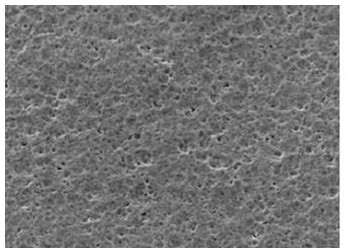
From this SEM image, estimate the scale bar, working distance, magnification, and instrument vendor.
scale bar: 50000 nm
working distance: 18.2 mm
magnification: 1 K X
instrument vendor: Hitachi